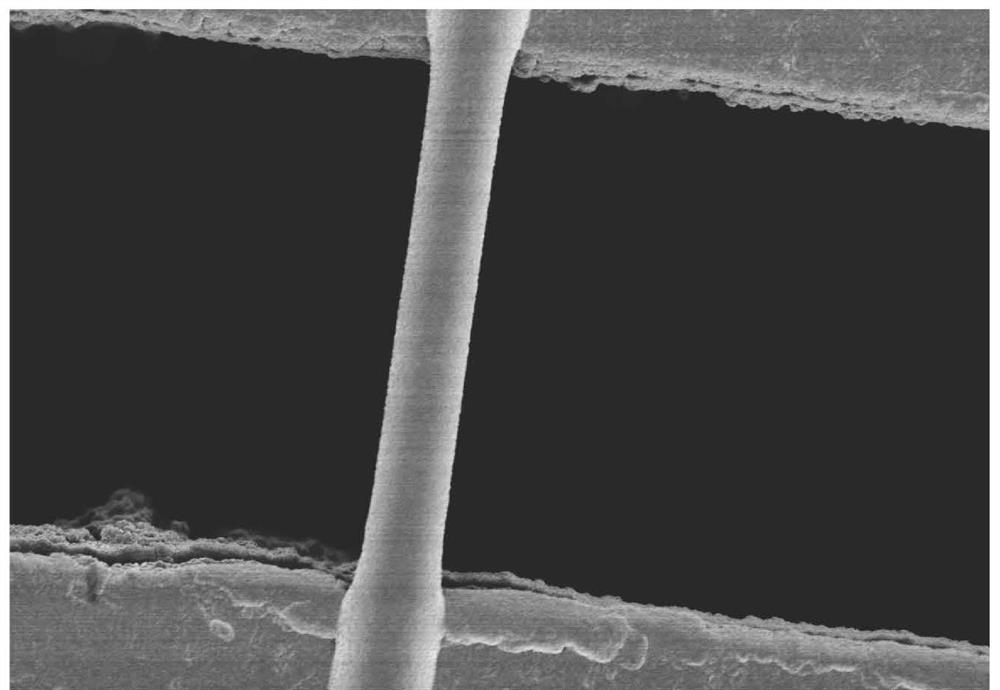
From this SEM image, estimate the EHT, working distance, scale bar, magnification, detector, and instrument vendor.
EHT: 1 kV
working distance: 2.5 mm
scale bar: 4000 nm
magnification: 13 K X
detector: SE(M)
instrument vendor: Hitachi